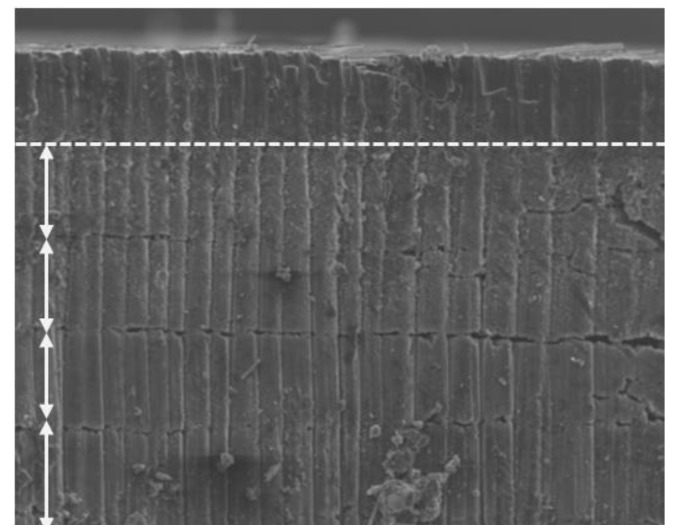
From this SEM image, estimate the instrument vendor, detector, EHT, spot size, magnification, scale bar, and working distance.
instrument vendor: FEI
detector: ETD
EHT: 20 kV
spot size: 7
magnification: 0.2 K X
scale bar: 500000 nm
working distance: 9.6 mm